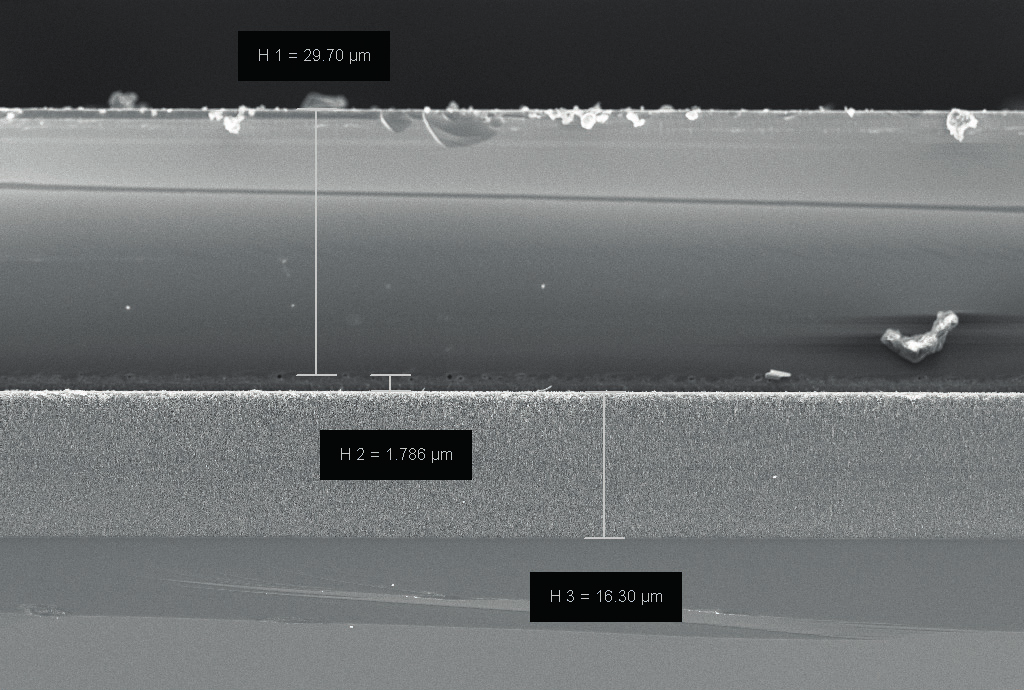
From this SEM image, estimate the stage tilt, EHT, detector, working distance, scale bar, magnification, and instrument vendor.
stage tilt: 0°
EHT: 5 kV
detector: InLens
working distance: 5.8 mm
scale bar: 10000 nm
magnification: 1 K X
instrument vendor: Zeiss